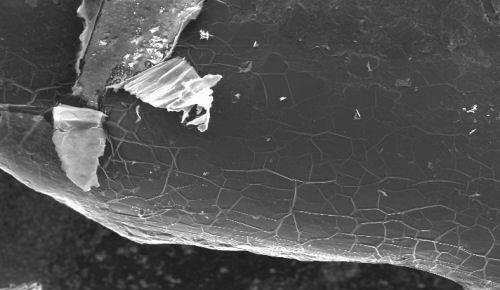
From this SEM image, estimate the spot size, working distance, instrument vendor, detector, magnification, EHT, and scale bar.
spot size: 5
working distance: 15.5 mm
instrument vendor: FEI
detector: SE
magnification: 0.062 K X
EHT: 30 kV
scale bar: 500000 nm